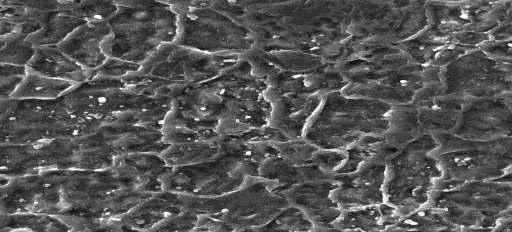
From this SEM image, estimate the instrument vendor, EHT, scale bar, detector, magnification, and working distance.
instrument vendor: Tescan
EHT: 25 kV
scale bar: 5000 nm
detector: SE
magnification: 5 K X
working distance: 18.684 mm